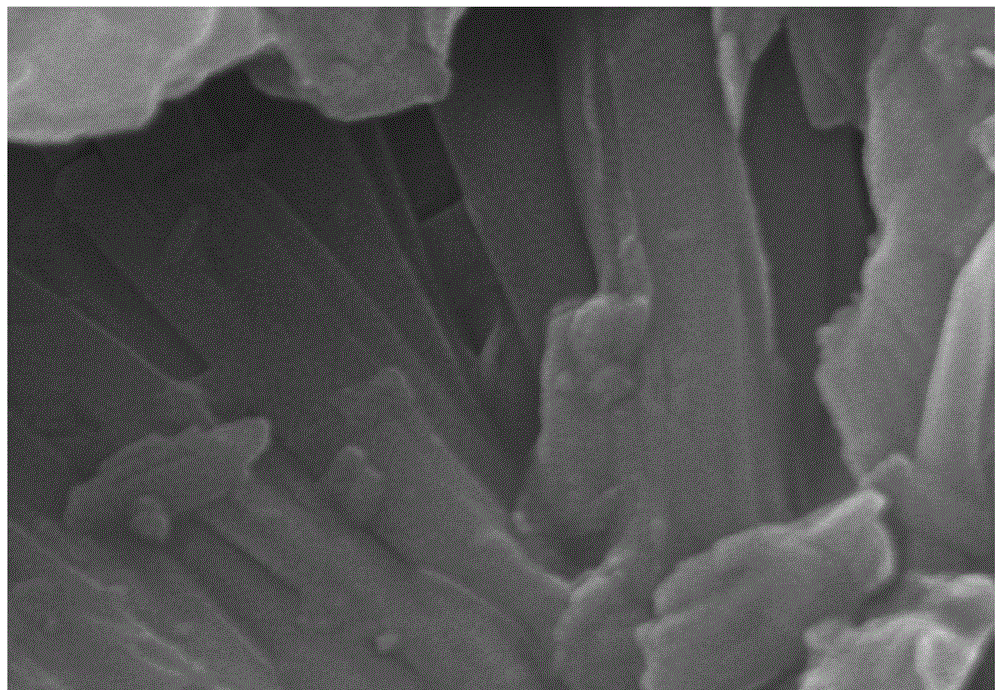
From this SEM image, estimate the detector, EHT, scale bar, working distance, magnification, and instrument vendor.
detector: SE(U)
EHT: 15 kV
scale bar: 500 nm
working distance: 11.7 mm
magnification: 60 K X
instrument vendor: Hitachi